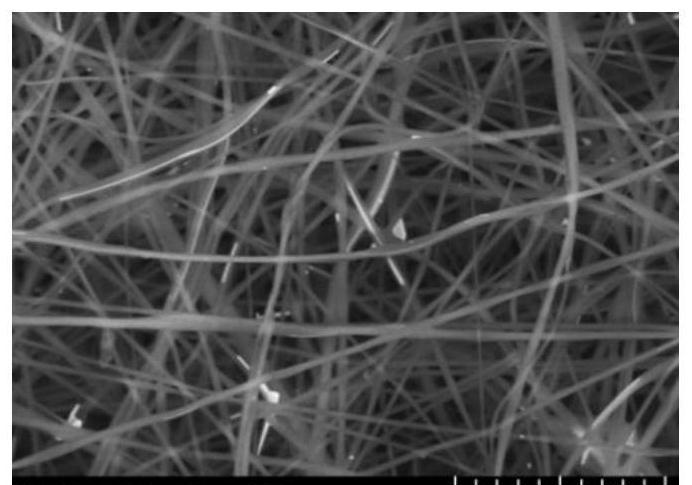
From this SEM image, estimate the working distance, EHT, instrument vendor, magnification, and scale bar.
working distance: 16.8 mm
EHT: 15 kV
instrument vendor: Hitachi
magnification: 2 K X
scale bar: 20000 nm